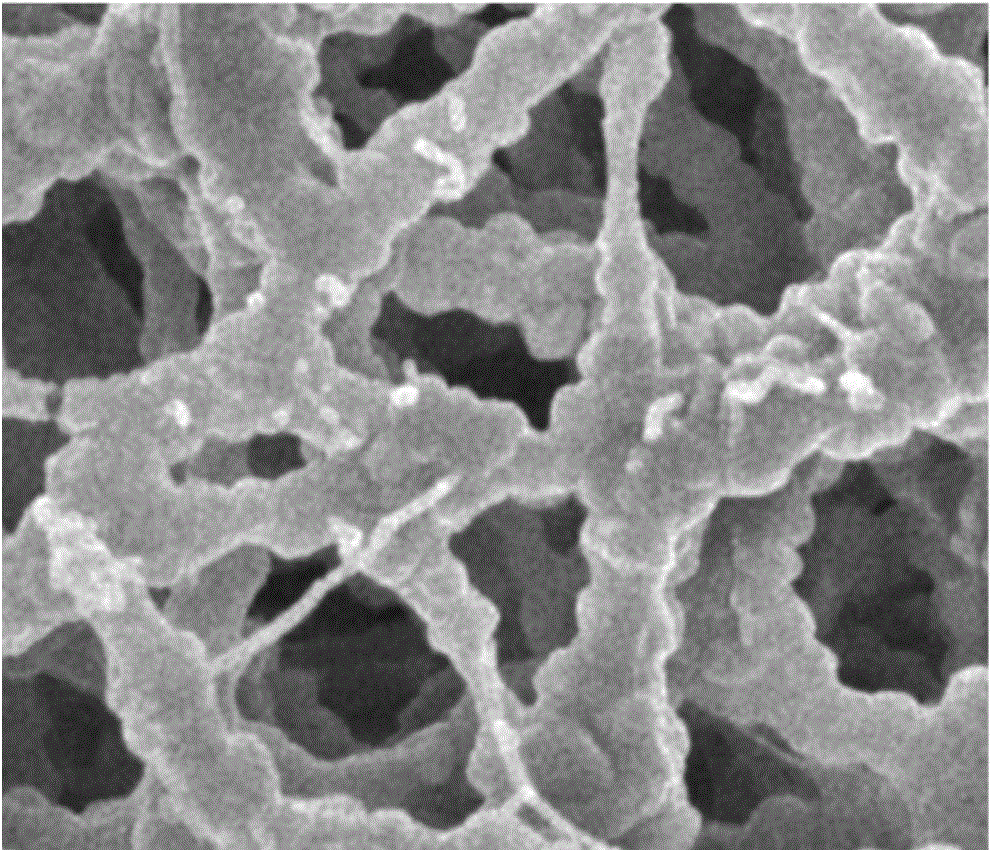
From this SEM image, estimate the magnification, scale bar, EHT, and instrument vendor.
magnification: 10 K X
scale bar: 1000 nm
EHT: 5 kV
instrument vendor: JEOL